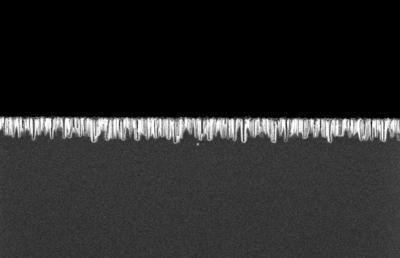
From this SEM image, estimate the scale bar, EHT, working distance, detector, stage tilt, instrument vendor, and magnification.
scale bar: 1000 nm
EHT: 3 kV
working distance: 4.3 mm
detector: InLens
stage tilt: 0°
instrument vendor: Zeiss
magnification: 10 K X